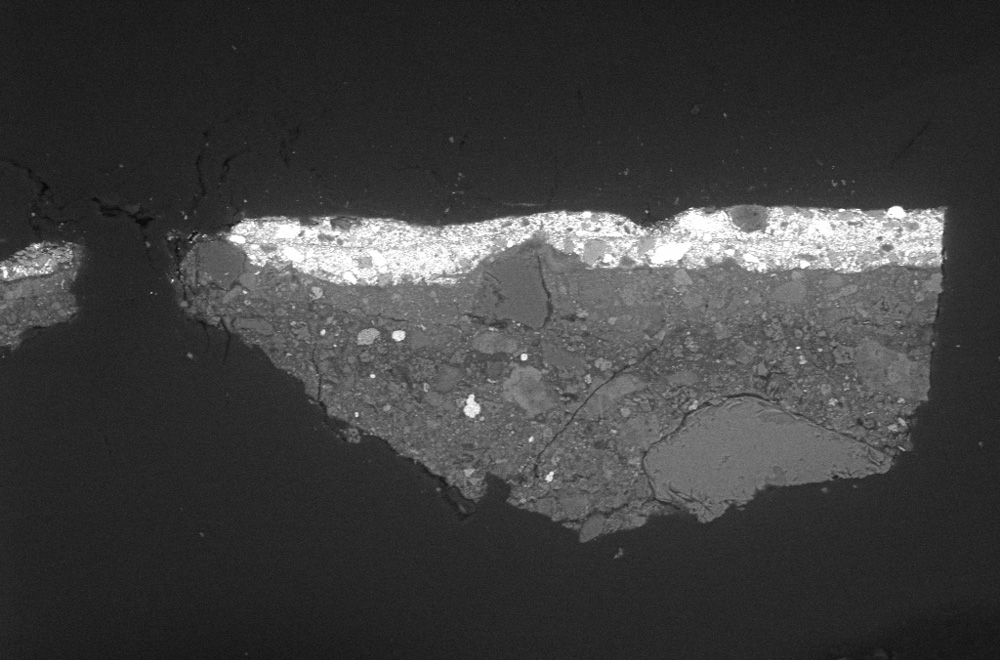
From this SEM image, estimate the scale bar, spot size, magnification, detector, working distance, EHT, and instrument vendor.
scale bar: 20000 nm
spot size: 380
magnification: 0.5 K X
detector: NTS BSD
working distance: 10 mm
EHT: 20 kV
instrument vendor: Zeiss